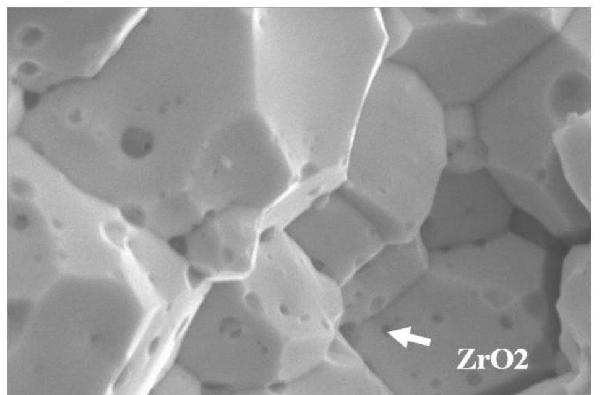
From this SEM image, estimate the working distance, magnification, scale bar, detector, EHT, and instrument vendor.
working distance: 8.7 mm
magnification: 15 K X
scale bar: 1000 nm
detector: SE2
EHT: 15 kV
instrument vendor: Zeiss